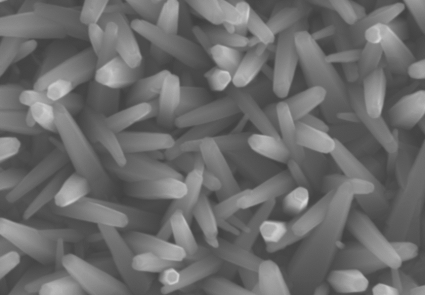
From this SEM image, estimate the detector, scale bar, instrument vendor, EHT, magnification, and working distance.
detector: SE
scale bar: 3000 nm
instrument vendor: Hitachi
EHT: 15 kV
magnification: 15 K X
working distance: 5.3 mm